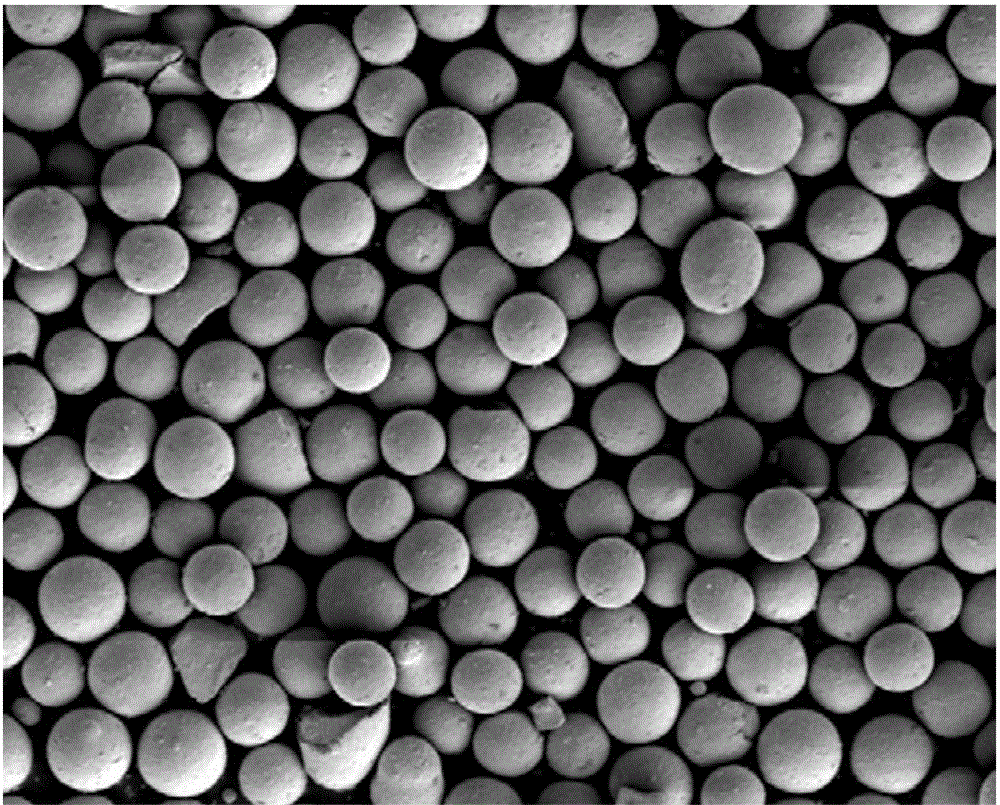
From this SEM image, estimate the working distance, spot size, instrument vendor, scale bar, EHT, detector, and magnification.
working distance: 4.7 mm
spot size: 3.5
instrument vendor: FEI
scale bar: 100000 nm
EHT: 5 kV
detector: ETD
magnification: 0.5 K X